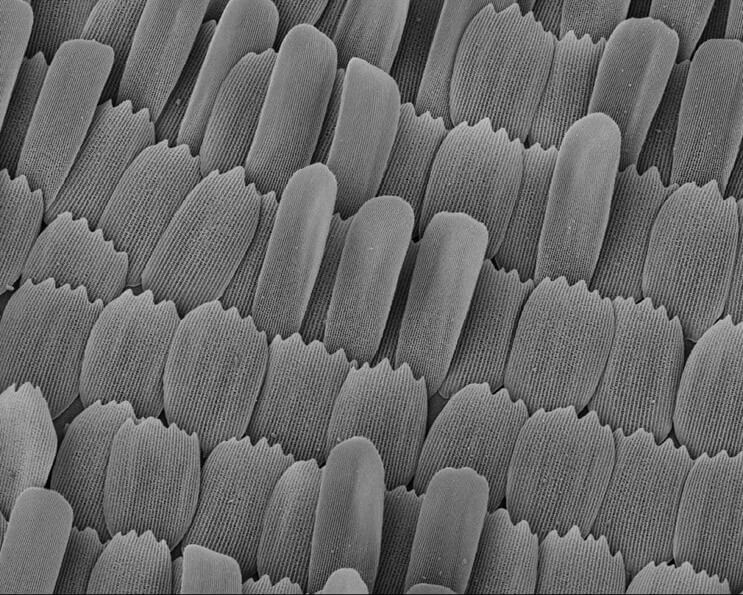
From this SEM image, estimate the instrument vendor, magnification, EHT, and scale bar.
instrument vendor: JEOL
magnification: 0.3 K X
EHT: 10 kV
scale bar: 100000 nm